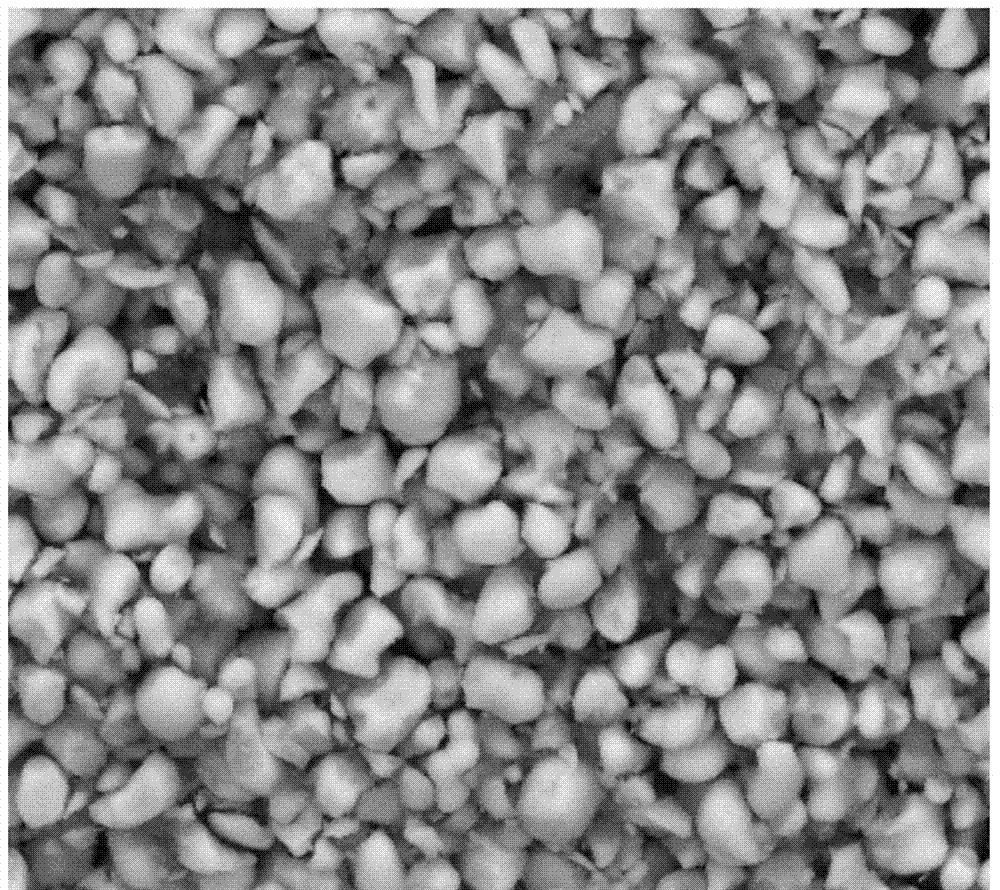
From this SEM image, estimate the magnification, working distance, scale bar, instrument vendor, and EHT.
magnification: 10 K X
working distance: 4.8 mm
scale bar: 5000 nm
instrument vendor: FEI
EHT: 20 kV